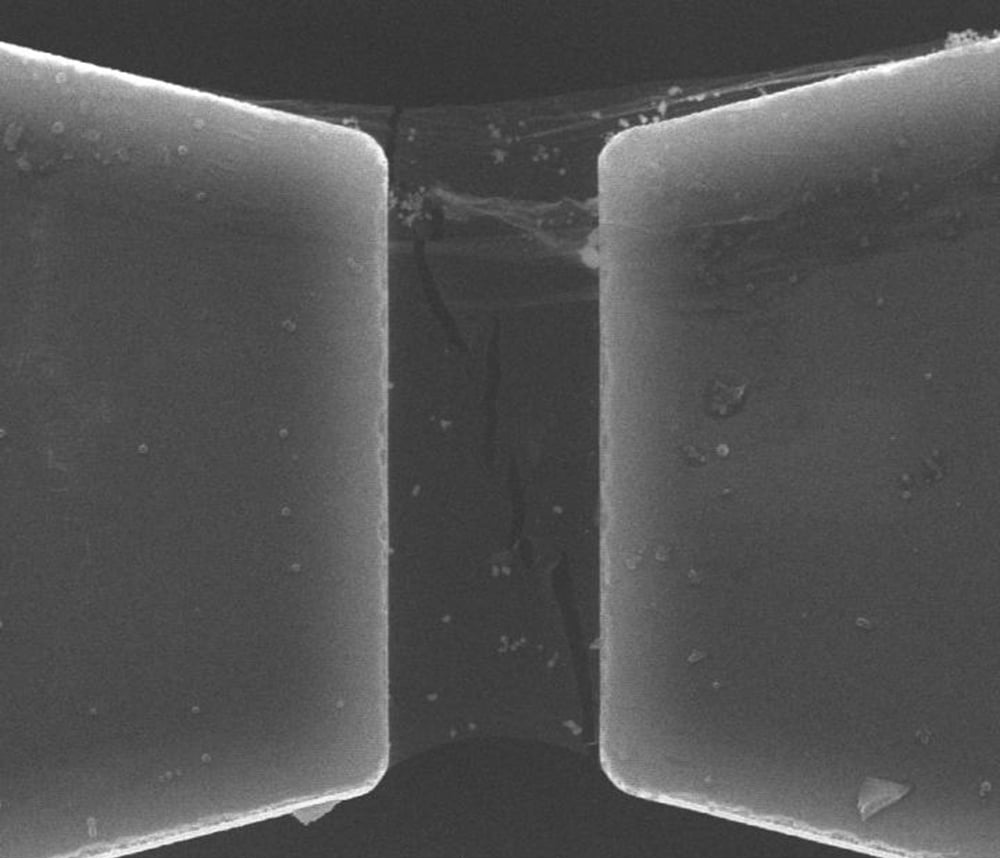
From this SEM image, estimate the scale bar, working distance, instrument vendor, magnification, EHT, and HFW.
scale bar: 10000 nm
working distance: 8.2 mm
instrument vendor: FEI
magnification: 10 K X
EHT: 20 kV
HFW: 29.8 µm